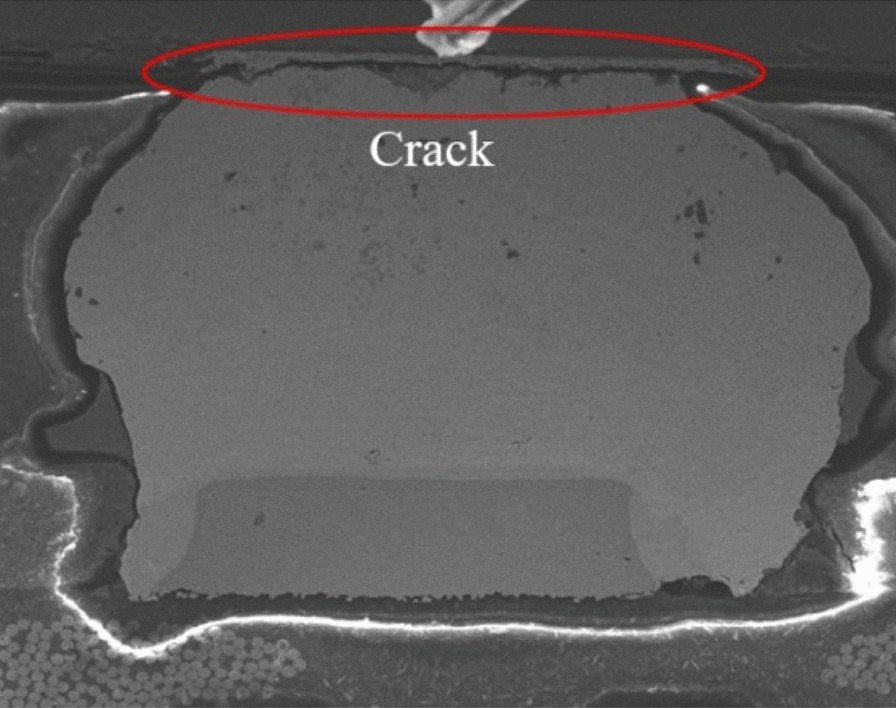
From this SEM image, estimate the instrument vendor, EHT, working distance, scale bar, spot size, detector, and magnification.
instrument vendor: FEI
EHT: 20 kV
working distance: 9.9 mm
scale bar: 100000 nm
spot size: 4.5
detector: ETD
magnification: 0.8 K X